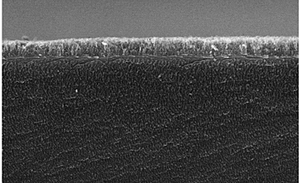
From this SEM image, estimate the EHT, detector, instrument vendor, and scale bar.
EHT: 15 kV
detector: InLens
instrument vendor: Zeiss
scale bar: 1000 nm